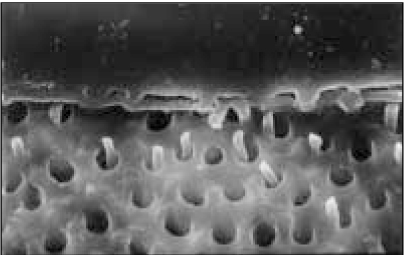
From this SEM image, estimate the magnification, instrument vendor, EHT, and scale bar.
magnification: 2 K X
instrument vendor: Hitachi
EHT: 20 kV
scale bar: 20000 nm